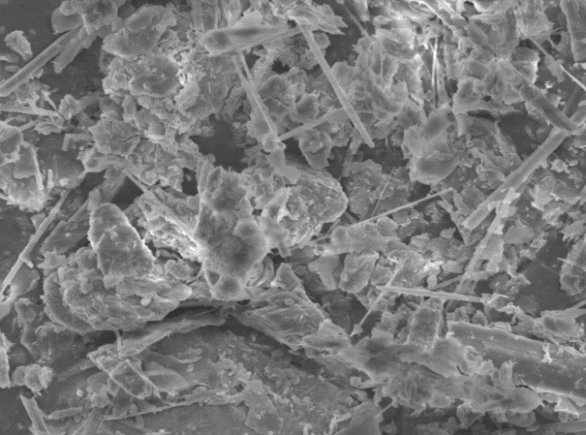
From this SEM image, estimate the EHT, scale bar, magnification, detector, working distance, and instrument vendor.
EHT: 5 kV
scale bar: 1000 nm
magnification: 5 K X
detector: SEI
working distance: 16.8 mm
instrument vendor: JEOL